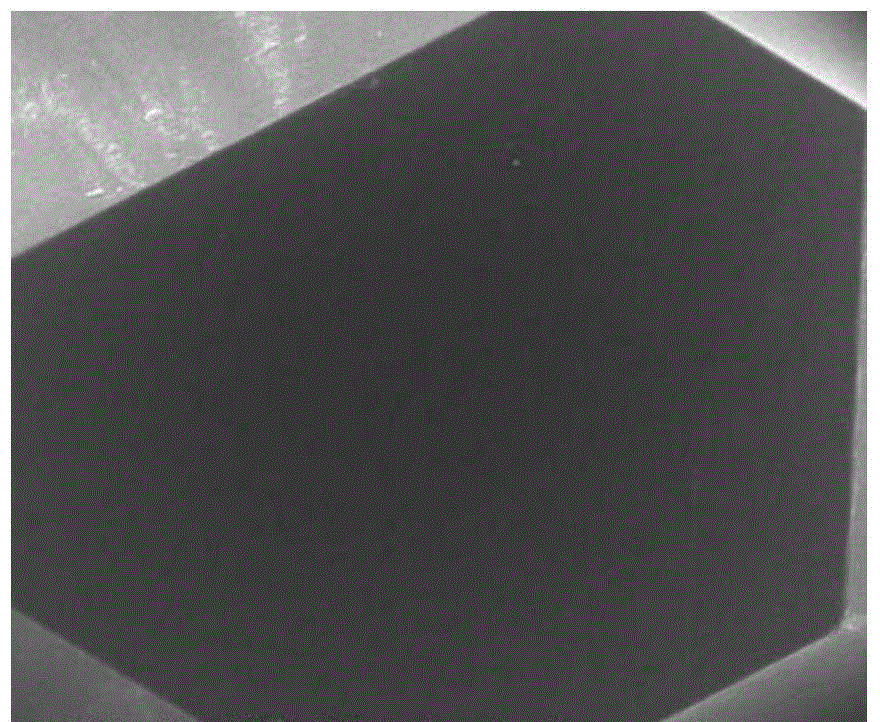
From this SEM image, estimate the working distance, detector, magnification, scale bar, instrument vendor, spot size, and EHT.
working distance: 11.9 mm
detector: ETD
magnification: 0.5 K X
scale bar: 100000 nm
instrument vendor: FEI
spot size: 3.5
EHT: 20 kV